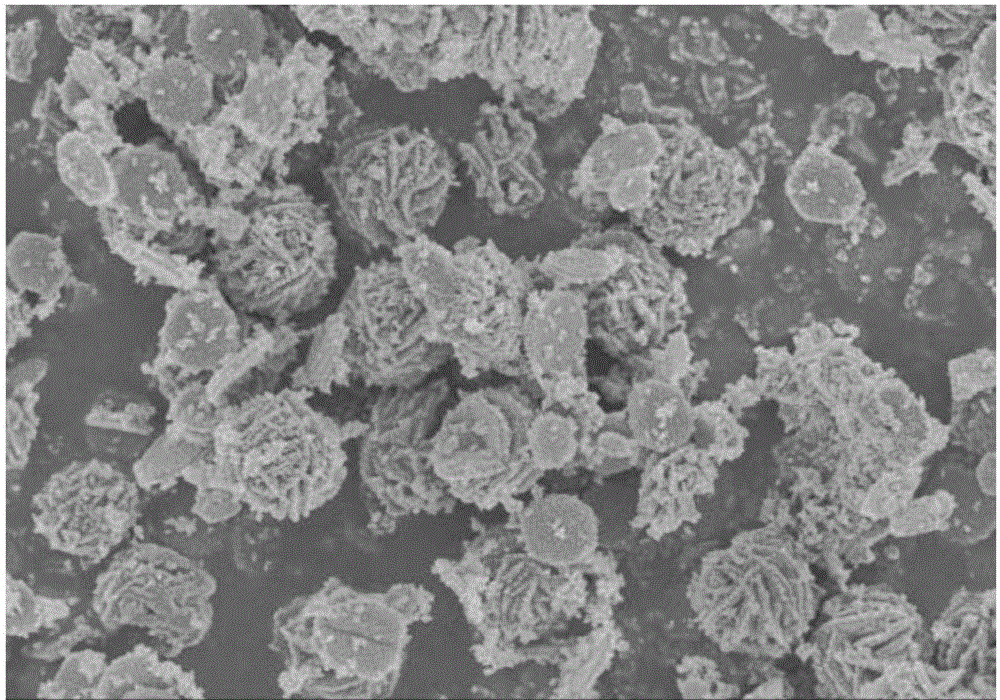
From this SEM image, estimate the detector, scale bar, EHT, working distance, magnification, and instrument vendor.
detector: SE(M)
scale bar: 5000 nm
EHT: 3 kV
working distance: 9.4 mm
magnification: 10 K X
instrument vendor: Hitachi